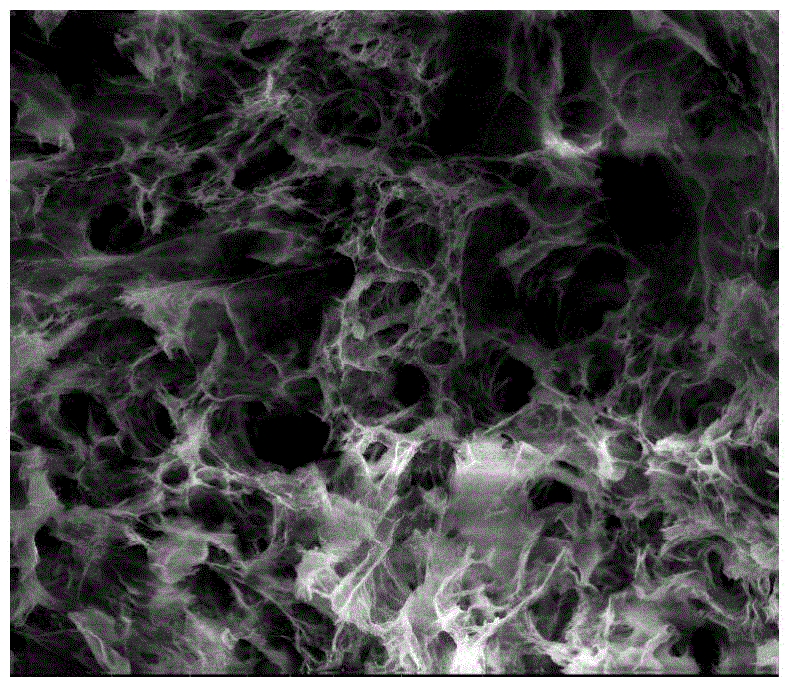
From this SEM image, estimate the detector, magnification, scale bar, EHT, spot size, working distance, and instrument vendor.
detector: ETD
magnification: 2 K X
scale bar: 50000 nm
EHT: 20 kV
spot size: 3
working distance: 10 mm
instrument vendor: FEI